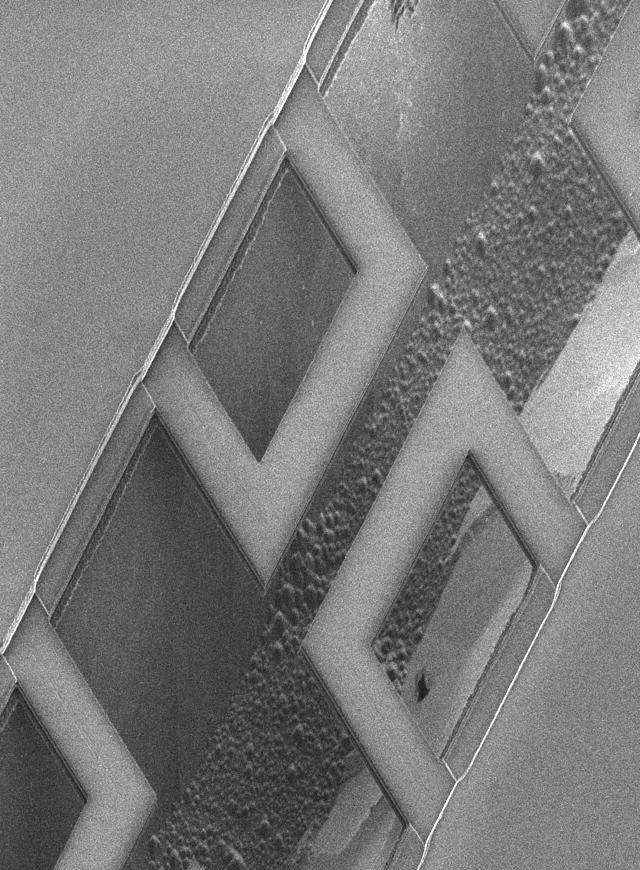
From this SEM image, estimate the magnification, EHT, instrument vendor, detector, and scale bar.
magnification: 1 K X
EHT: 3 kV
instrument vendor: JEOL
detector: SEI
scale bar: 10000 nm